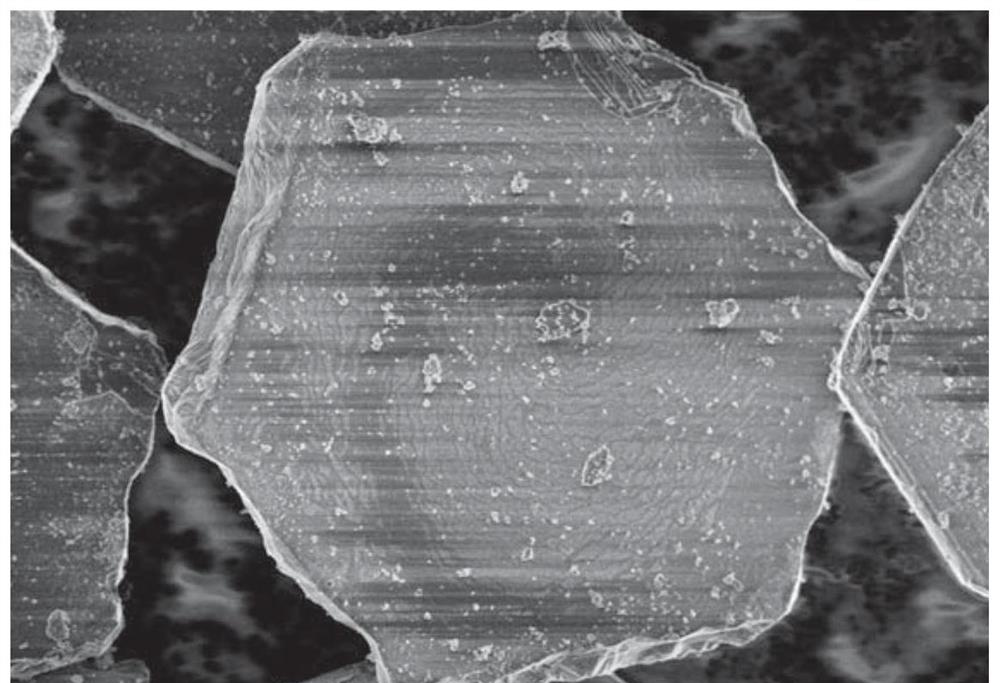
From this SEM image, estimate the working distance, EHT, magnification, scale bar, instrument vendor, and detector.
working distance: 8.3 mm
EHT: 2 kV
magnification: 3 K X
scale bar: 10000 nm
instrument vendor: Hitachi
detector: SE(UL)